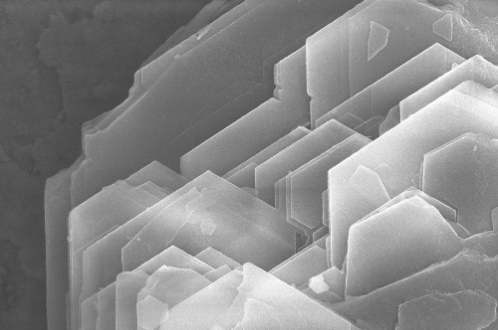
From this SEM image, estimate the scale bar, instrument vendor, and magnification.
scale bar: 5000 nm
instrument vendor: Hitachi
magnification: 10 K X